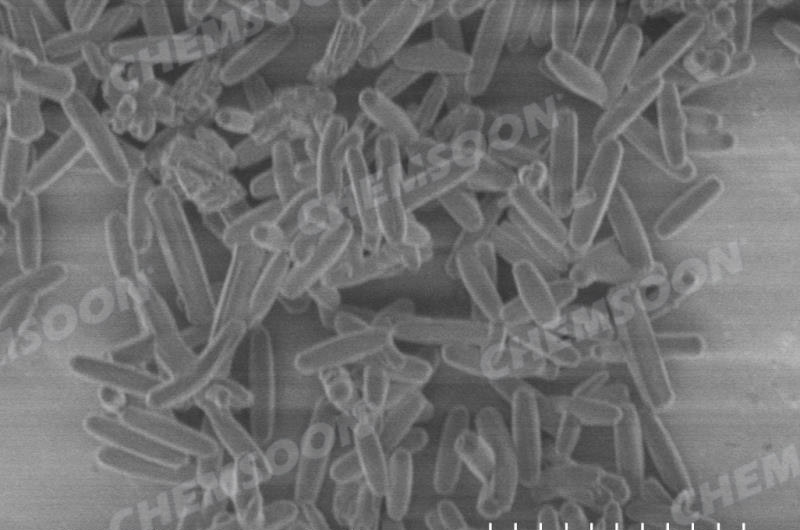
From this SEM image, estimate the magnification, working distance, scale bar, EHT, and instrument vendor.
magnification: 8 K X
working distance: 8 mm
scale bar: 5000 nm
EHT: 1 kV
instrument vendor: Hitachi